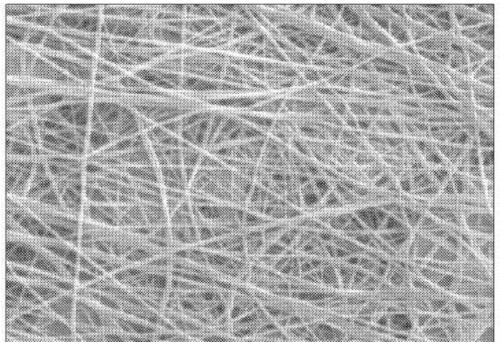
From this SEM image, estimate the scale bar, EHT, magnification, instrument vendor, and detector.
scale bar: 10000 nm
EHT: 30 kV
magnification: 10 K X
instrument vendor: other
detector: SE